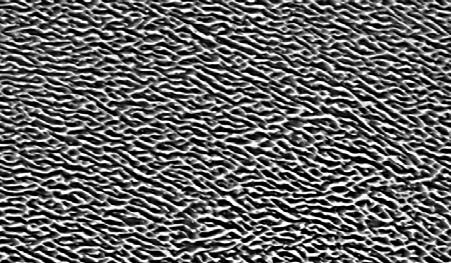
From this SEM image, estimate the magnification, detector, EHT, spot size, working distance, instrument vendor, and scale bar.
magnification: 2.5 K X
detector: BSE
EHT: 20 kV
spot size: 4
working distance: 7.6 mm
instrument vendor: FEI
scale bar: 10000 nm